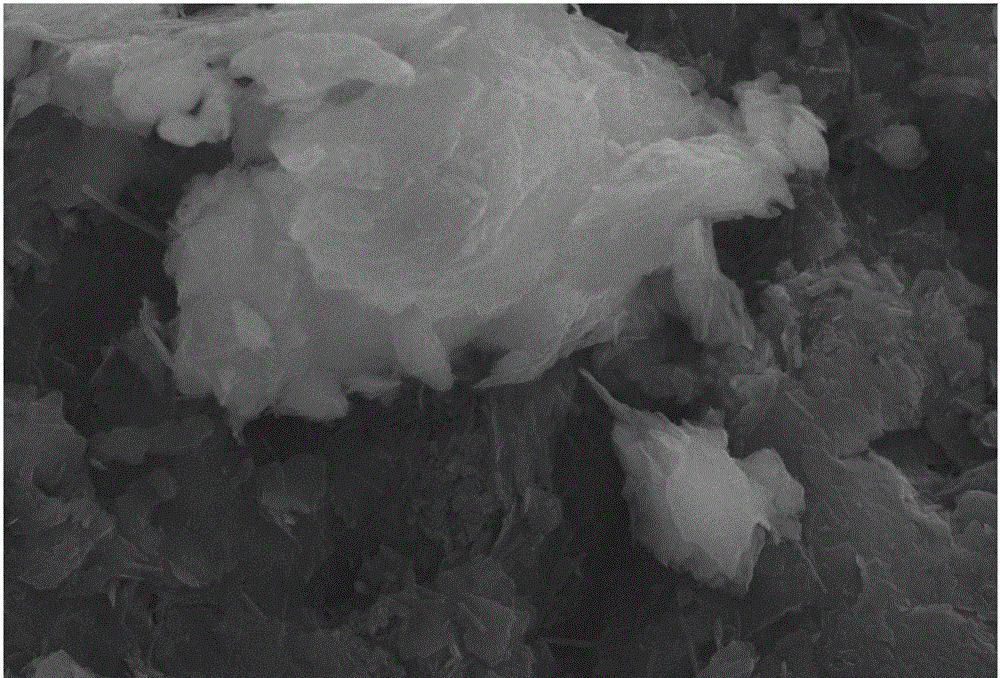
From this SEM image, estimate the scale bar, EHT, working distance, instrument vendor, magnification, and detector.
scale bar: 5000 nm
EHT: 30 kV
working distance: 7 mm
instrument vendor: JEOL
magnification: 3 K X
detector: SEI